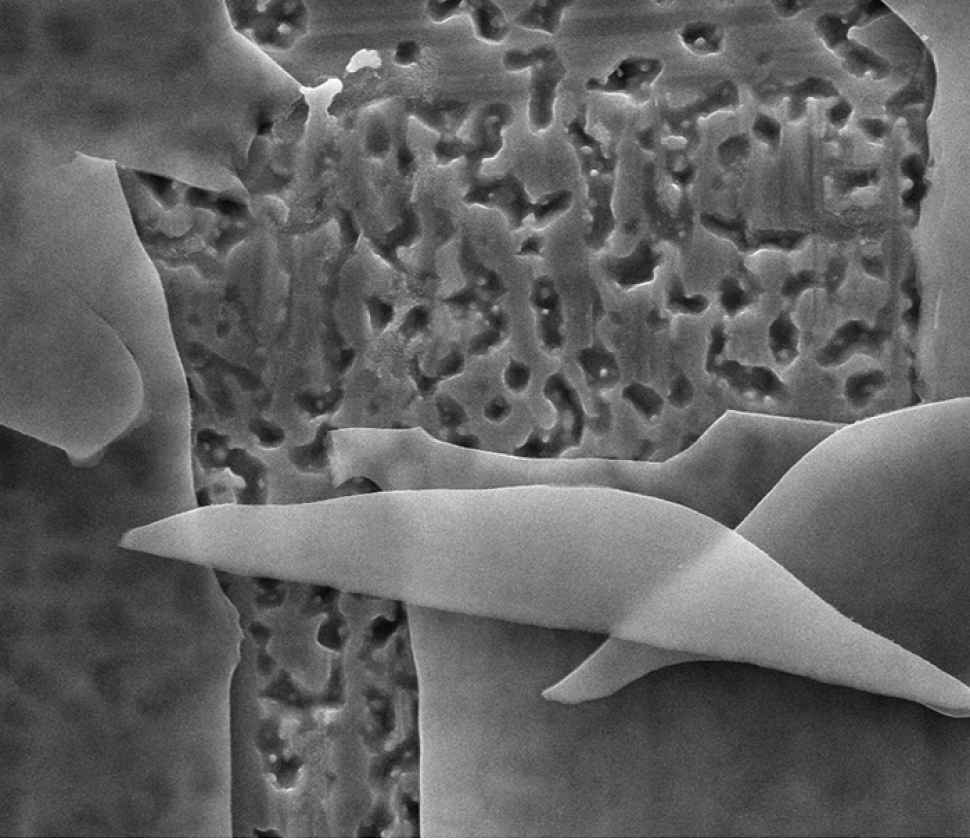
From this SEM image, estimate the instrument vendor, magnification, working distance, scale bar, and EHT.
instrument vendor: FEI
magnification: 12 K X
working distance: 10.2 mm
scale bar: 10000 nm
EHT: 20 kV